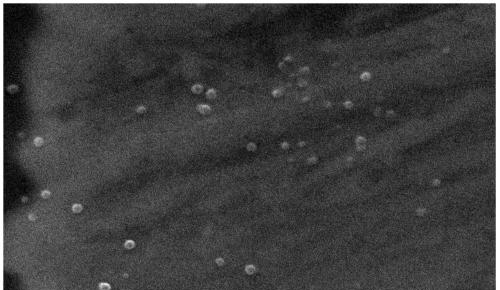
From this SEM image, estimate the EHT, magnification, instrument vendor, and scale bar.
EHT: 20 kV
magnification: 20 K X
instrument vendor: JEOL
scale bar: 1000 nm